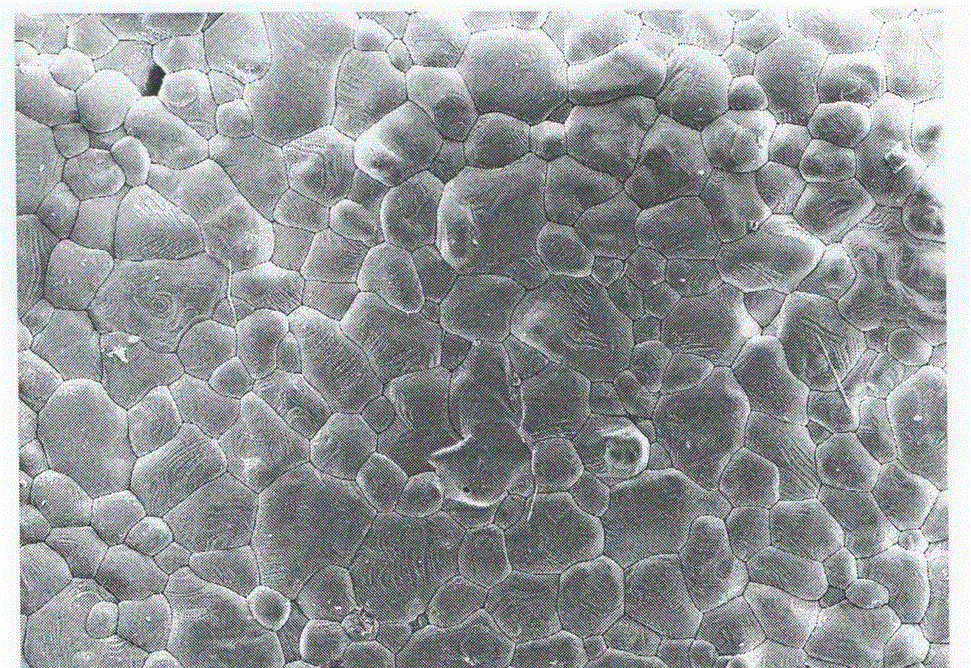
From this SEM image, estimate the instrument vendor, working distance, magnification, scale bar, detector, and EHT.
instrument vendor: Zeiss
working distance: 9.7 mm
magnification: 1 K X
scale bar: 10000 nm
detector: SE2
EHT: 10 kV